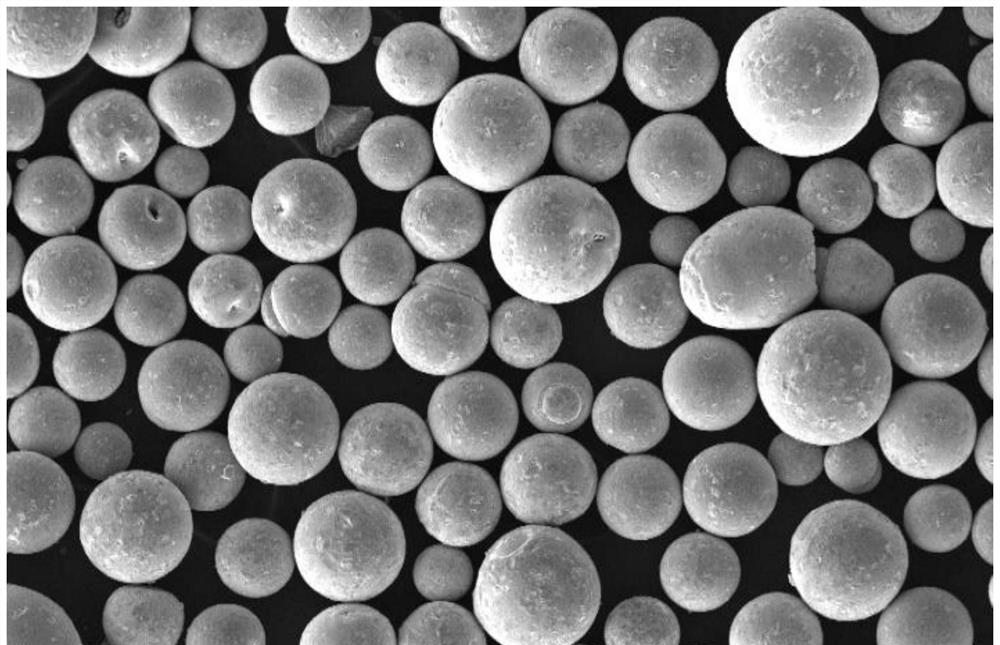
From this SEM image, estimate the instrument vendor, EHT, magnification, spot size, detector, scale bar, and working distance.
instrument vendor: FEI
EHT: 10 kV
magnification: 0.2 K X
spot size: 2.5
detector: SE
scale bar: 100000 nm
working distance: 7.9 mm